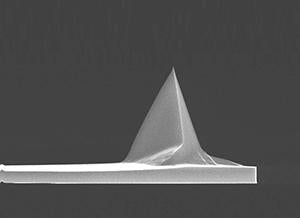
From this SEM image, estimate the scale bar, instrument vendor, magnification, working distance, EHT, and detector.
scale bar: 10000 nm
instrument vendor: FEI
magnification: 3.5 K X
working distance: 5 mm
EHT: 15 kV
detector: TLD